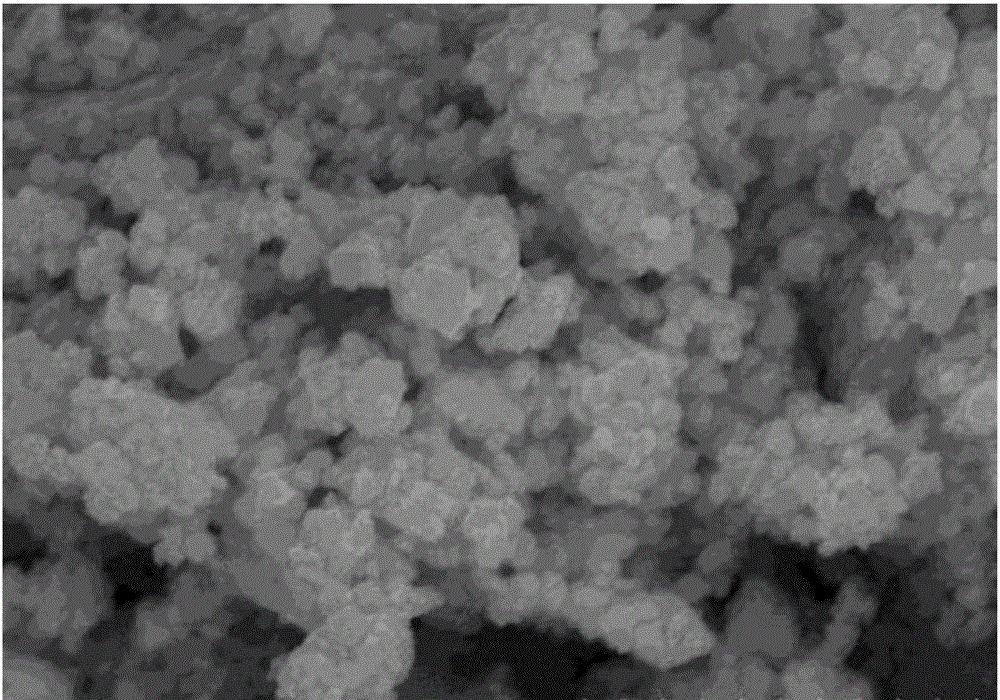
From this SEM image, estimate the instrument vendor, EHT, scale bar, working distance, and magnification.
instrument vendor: Hitachi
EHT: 15 kV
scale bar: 1000 nm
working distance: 9 mm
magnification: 50 K X